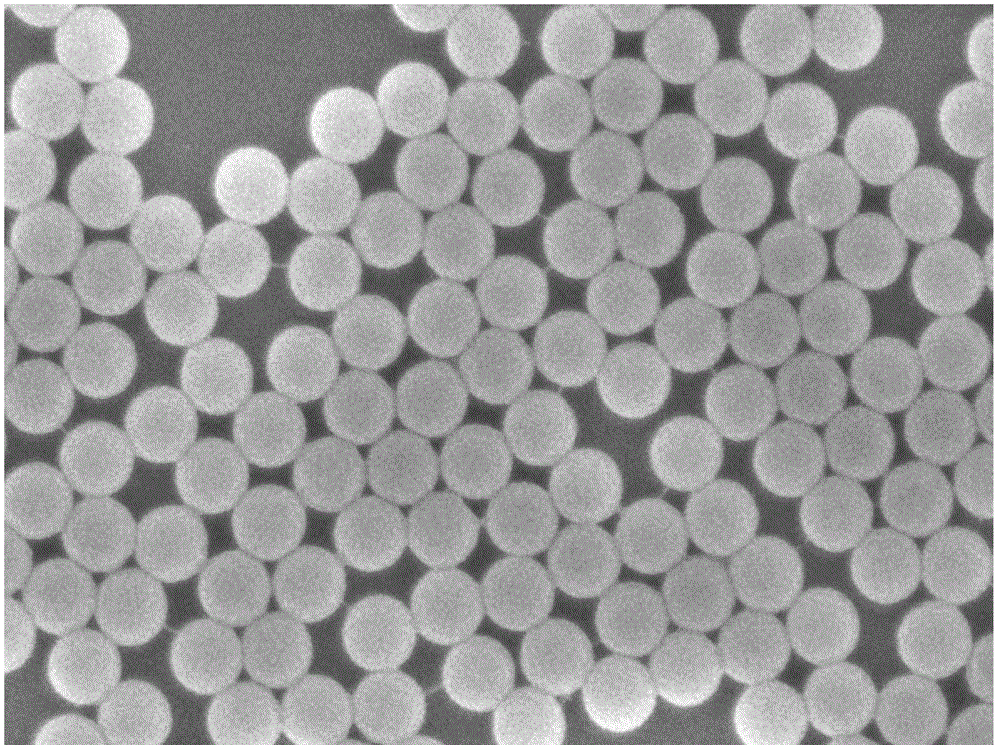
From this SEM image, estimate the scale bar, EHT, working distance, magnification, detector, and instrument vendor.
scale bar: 100 nm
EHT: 5 kV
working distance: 7.2 mm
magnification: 30 K X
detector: SEI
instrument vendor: JEOL